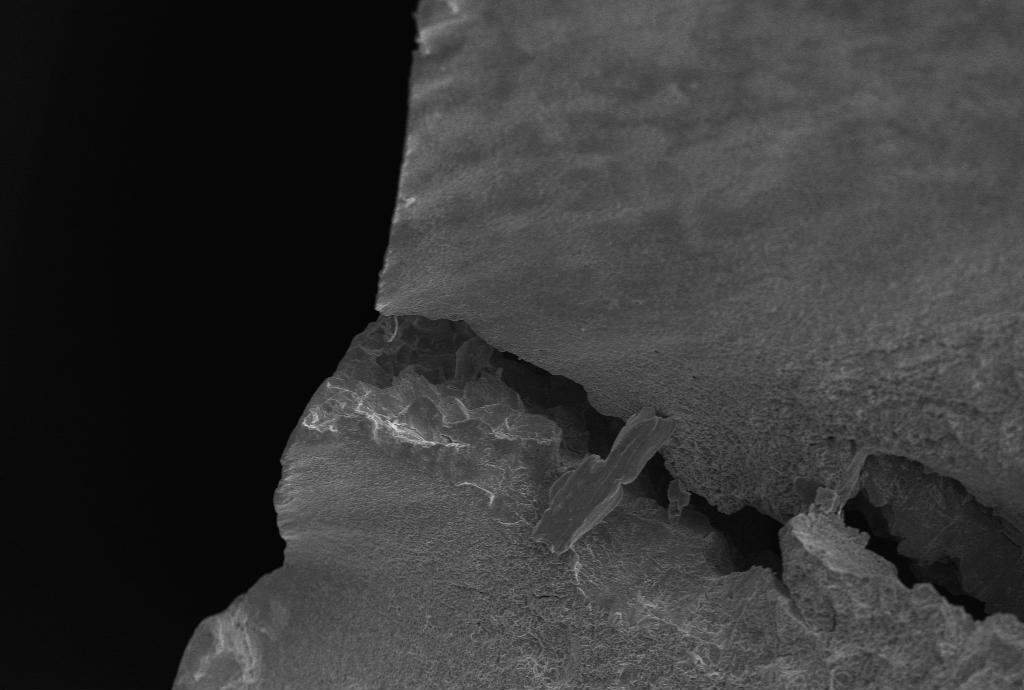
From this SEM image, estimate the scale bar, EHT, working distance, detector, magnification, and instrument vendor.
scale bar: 20000 nm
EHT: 20 kV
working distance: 18.5 mm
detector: SE1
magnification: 0.2 K X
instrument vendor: Zeiss